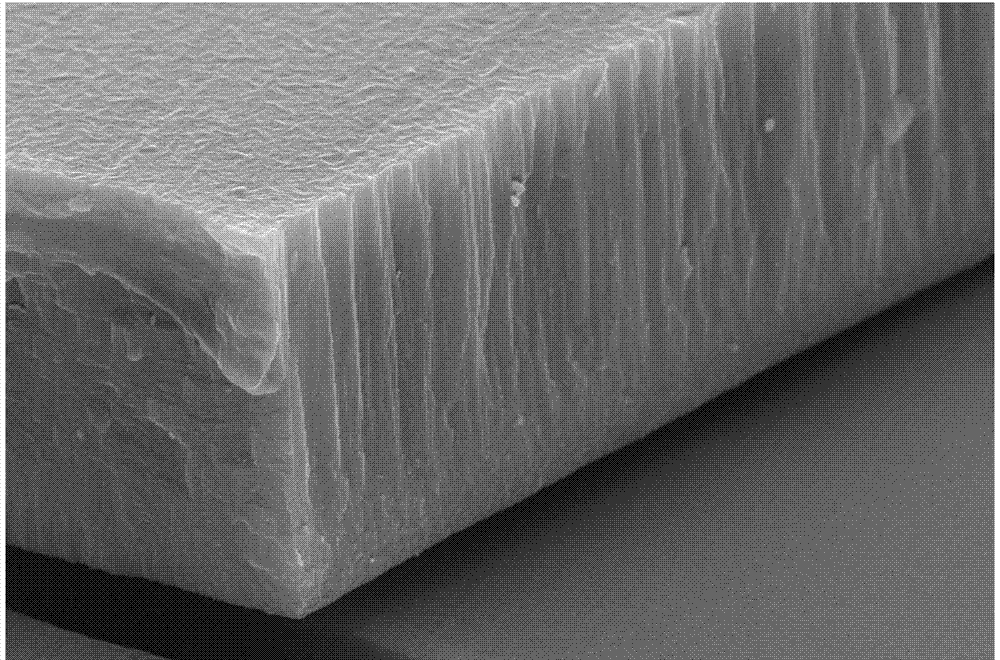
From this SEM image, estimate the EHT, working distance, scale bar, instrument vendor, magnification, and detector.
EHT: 15 kV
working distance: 5.7 mm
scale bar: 1000 nm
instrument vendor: Zeiss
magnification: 10 K X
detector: SE2